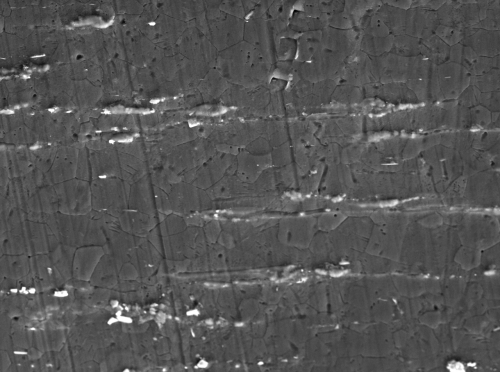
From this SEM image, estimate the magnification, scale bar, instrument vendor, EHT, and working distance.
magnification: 1 K X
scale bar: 10000 nm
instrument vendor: JEOL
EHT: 15 kV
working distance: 11.9 mm